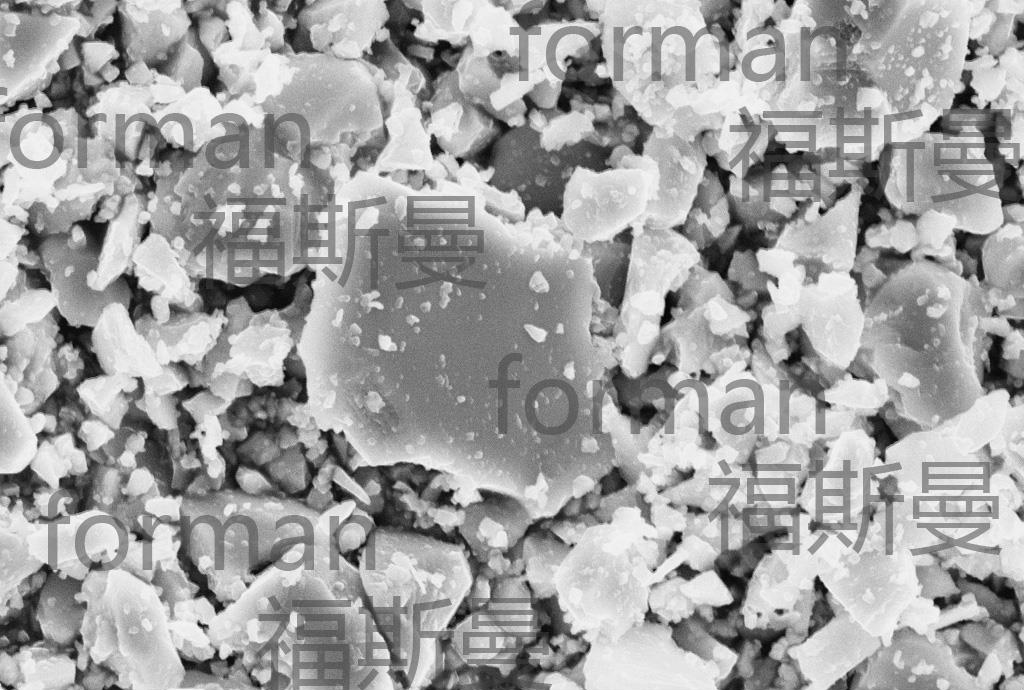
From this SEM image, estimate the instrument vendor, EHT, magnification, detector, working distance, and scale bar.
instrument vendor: Zeiss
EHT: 20 kV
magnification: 7 K X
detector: SE1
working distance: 21 mm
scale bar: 2000 nm